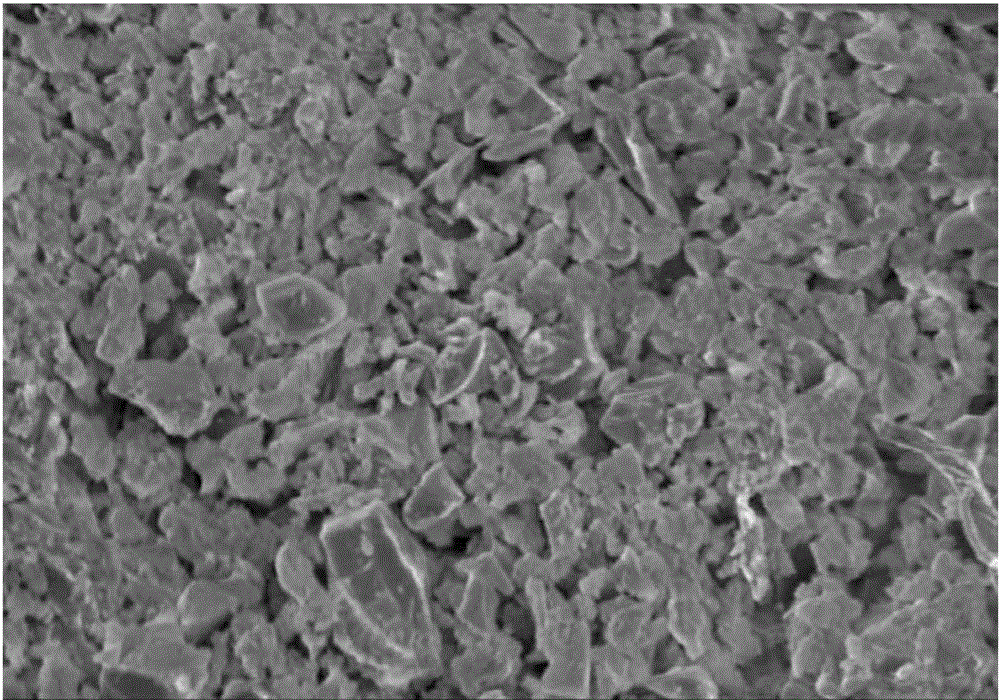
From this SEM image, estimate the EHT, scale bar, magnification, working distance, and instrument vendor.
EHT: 5 kV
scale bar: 10000 nm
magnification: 5 K X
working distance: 14.1 mm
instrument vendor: Hitachi